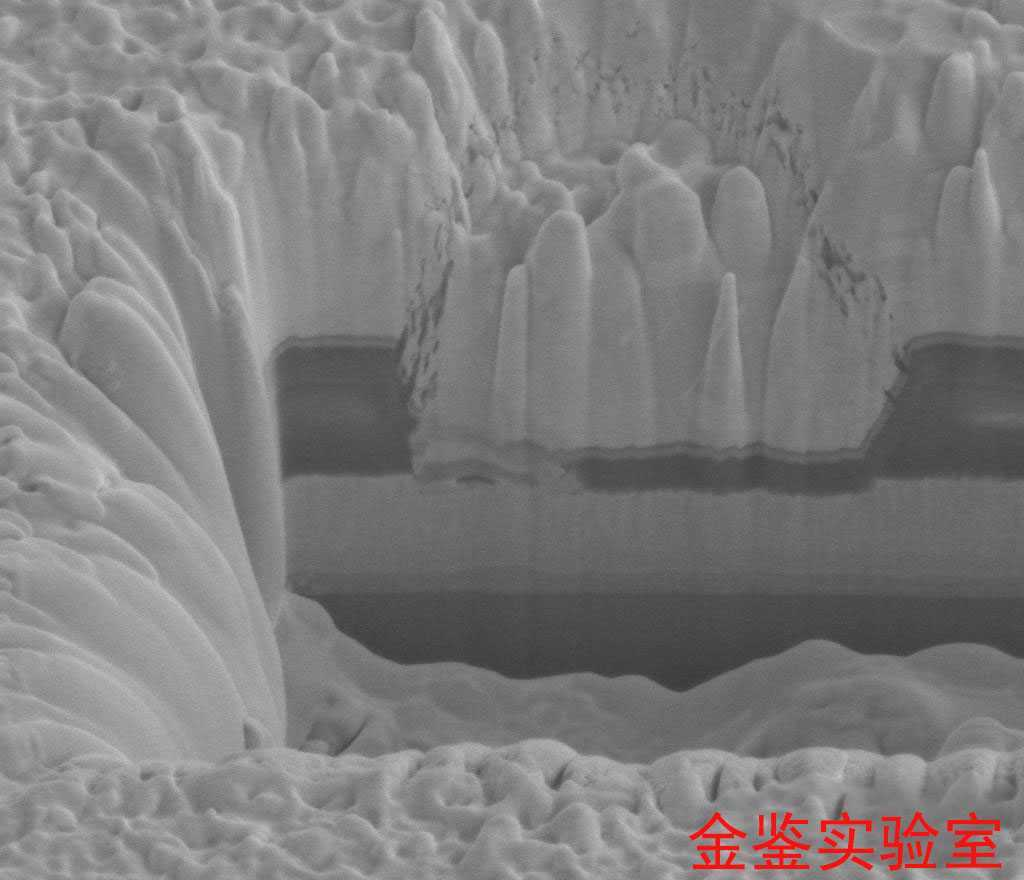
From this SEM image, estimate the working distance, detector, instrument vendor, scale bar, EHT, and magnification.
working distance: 4.9 mm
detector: ETD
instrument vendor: FEI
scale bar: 2000 nm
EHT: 5 kV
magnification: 40 K X